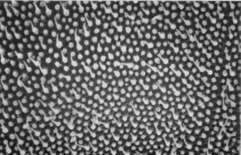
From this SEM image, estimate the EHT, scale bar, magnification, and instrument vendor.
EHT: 20 kV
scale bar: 1000 nm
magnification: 10 K X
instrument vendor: JEOL